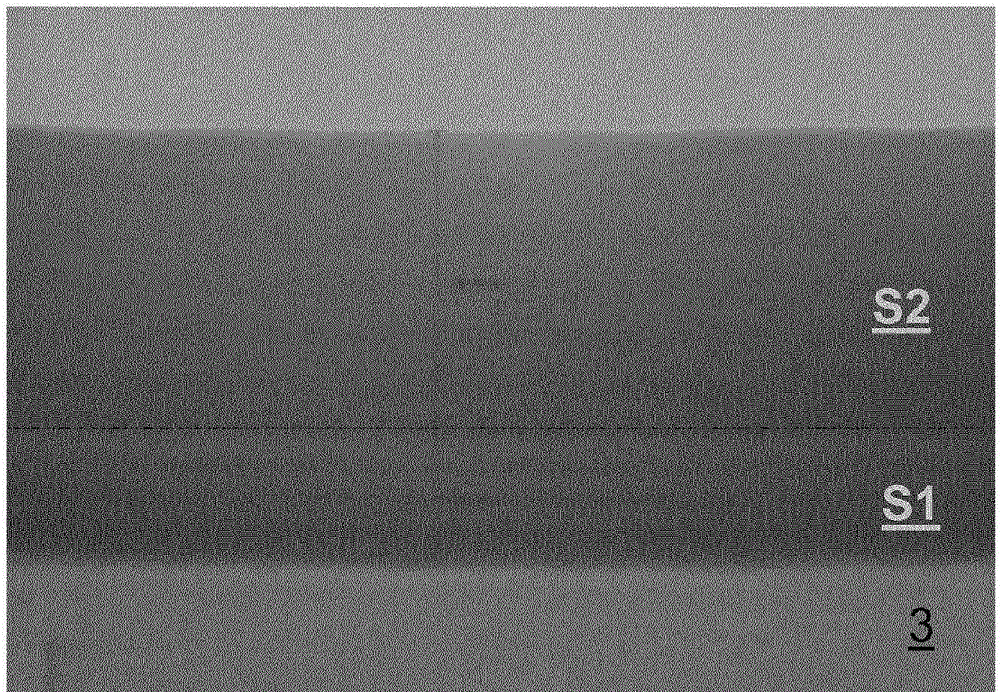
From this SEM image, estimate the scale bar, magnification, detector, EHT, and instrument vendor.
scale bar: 60 nm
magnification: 450 K X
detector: TE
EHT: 200 kV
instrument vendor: Hitachi